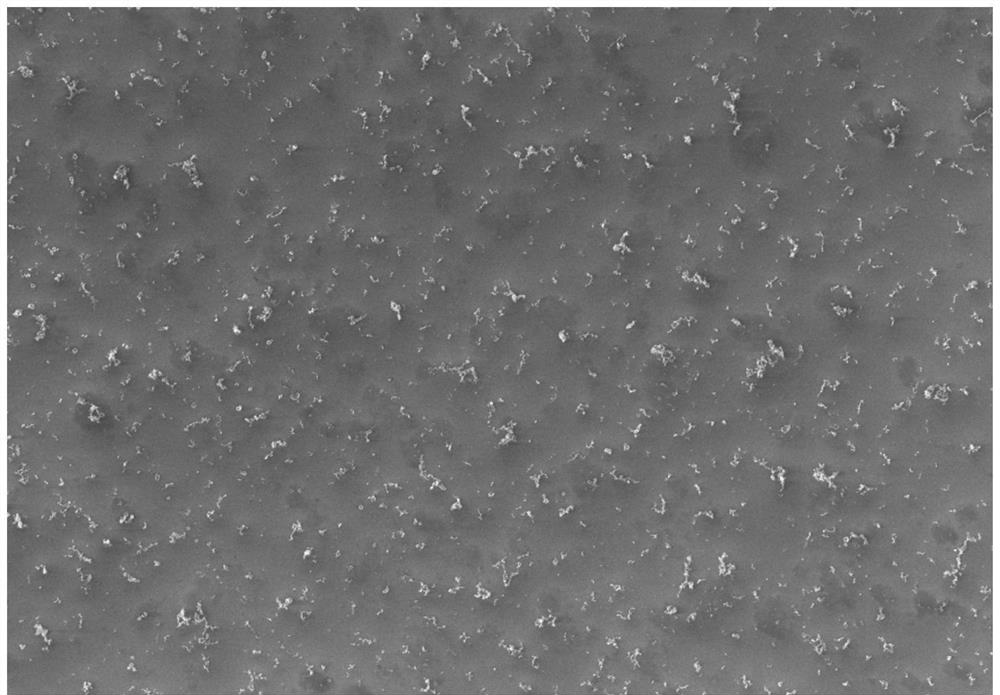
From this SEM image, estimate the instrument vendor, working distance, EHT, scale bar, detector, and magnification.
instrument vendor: Zeiss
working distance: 5 mm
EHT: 20 kV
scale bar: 1000 nm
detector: InLens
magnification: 5 K X